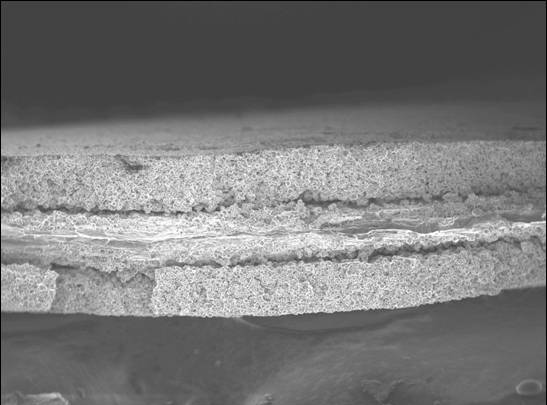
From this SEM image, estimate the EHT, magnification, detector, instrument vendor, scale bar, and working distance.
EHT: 5 kV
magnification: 0.25 K X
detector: SEI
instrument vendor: JEOL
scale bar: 100000 nm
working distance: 8.4 mm